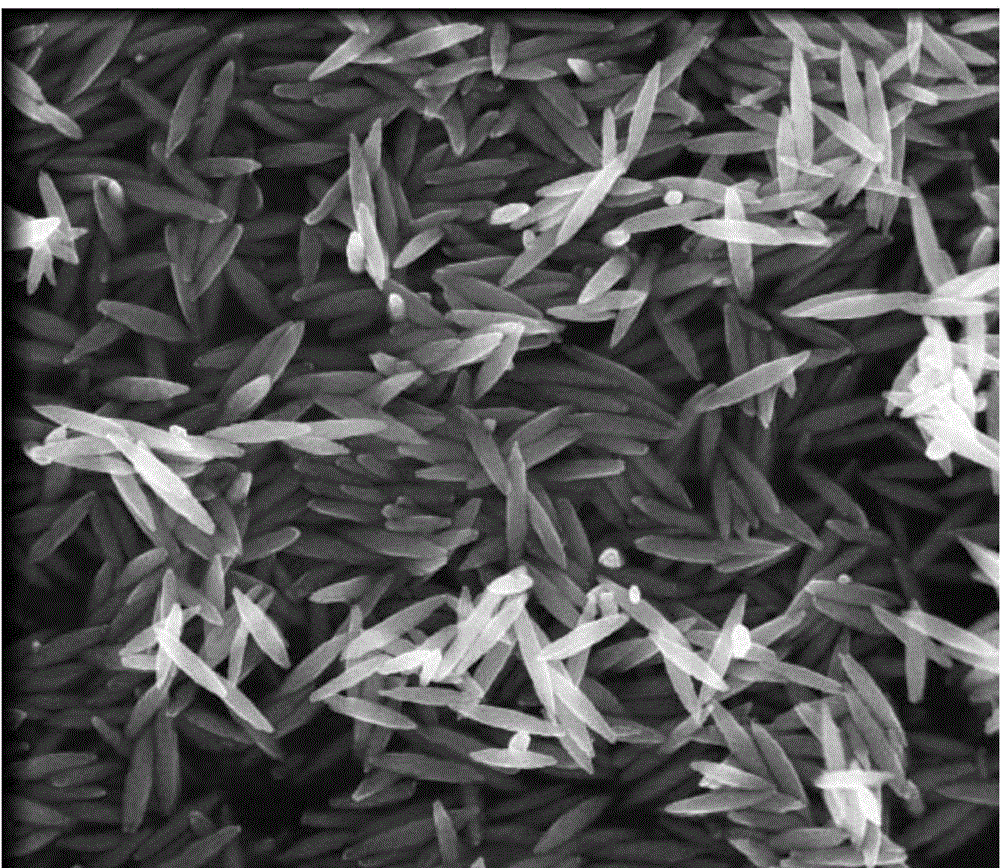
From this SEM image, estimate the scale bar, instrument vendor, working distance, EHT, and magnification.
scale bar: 1000 nm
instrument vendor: FEI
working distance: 4.9 mm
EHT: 10 kV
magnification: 80 K X